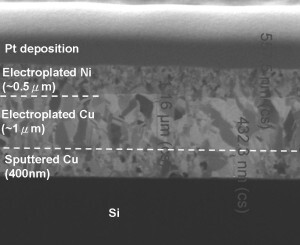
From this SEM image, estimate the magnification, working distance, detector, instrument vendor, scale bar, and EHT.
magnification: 65 K X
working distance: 19 mm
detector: ETD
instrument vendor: FEI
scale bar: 1000 nm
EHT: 30 kV